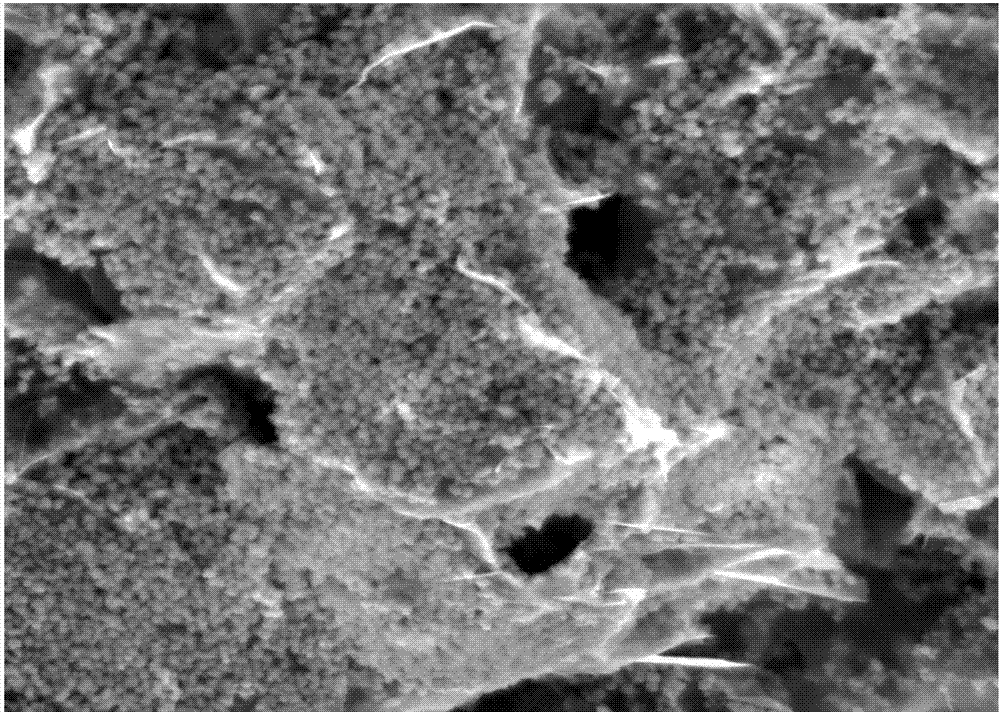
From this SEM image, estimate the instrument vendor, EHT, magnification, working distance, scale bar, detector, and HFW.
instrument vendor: FEI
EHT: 10 kV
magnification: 5 K X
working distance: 11 mm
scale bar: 10000 nm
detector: ETD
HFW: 25.4 µm